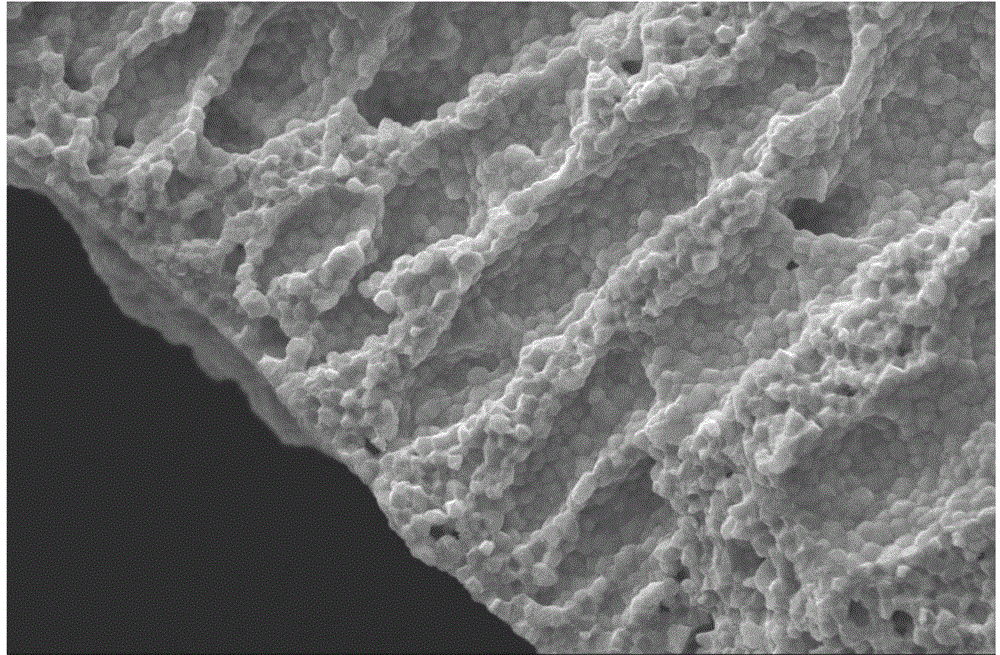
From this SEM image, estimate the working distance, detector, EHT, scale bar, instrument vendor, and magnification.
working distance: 8 mm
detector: SE1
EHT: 10 kV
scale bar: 2000 nm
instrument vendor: Zeiss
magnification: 6 K X